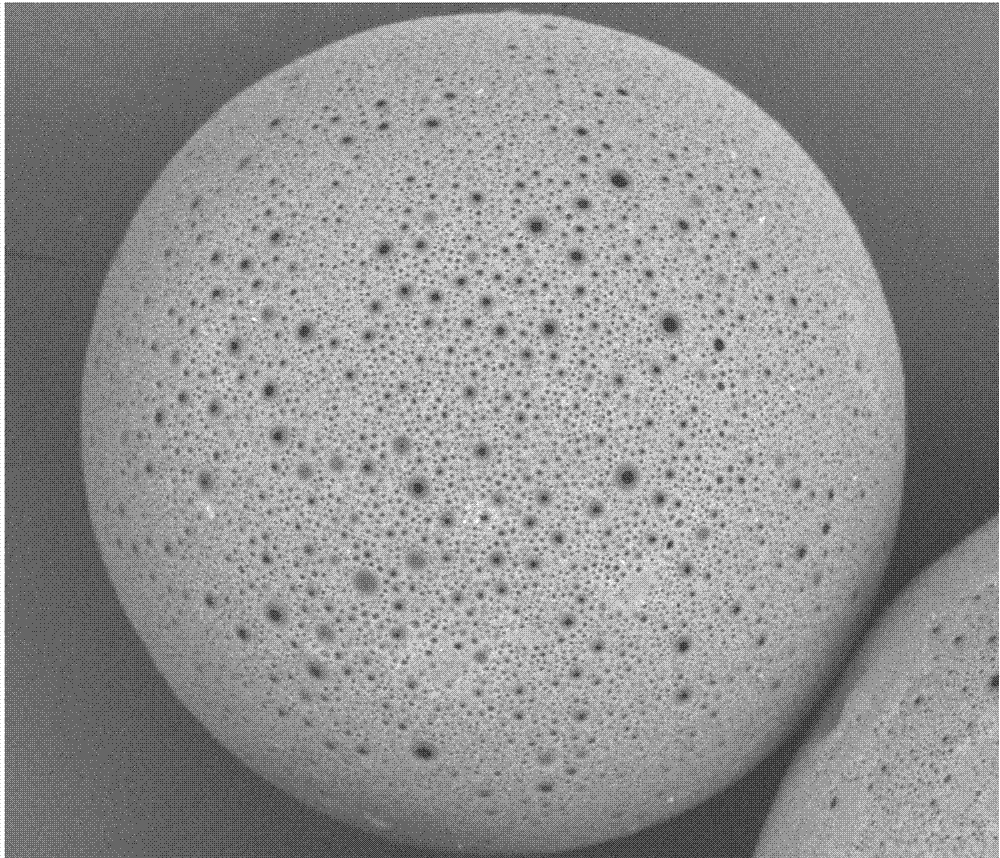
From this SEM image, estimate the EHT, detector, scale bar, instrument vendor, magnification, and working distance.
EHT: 15 kV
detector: DualBSD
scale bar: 100000 nm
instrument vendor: FEI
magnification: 1 K X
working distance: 11.7 mm